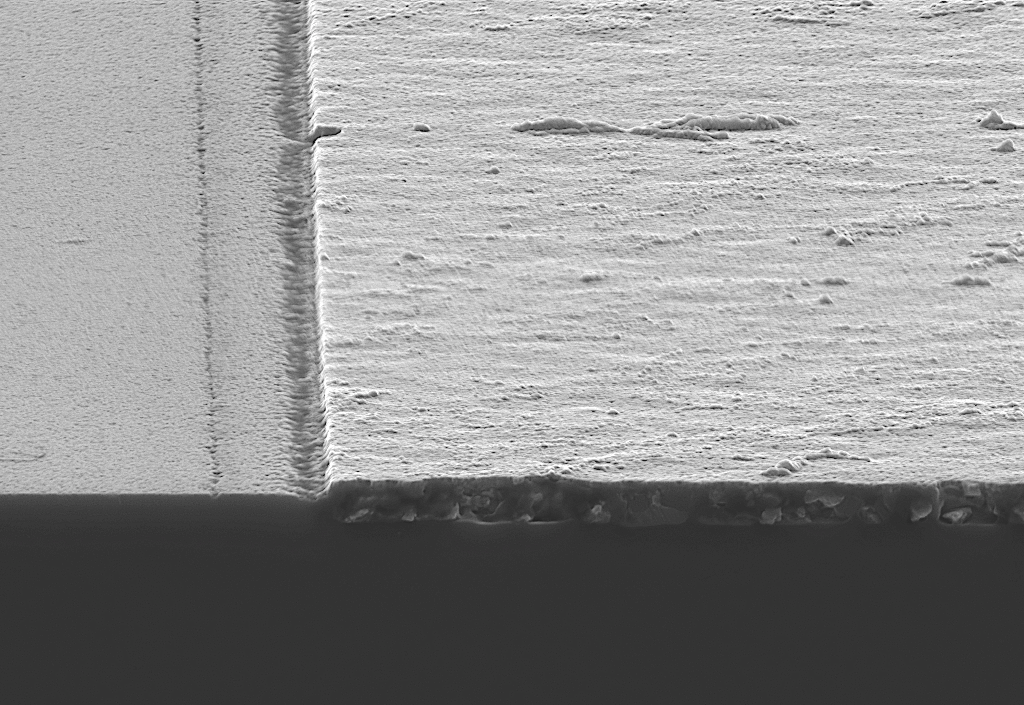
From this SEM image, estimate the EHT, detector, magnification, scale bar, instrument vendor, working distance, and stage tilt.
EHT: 7 kV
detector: InLens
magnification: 50 K X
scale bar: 200 nm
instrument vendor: Zeiss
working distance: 2 mm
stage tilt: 7.1°